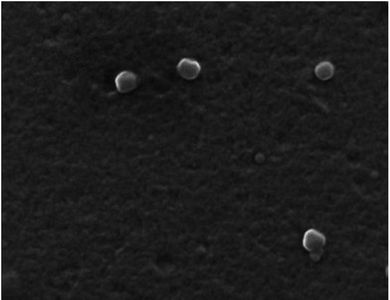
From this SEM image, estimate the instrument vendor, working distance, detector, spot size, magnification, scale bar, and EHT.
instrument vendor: FEI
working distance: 10.7 mm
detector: ETD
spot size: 3.5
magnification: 128.839 K X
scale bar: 500 nm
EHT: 15 kV